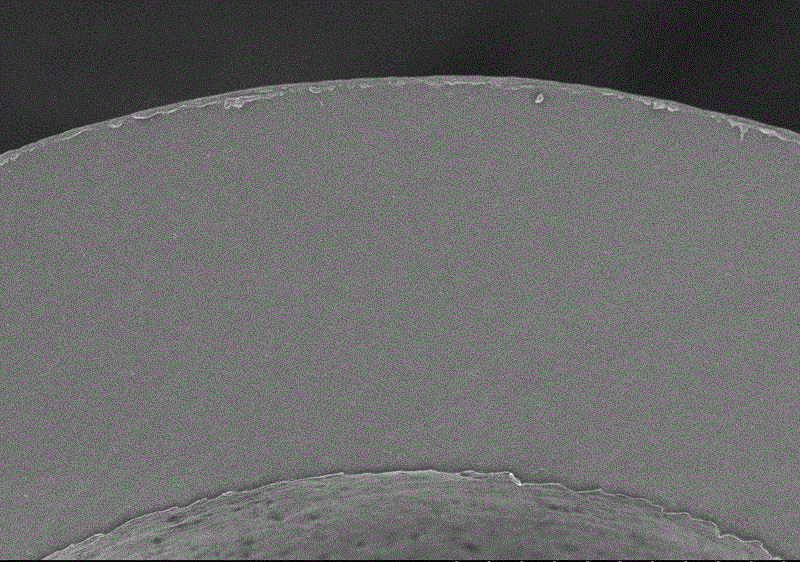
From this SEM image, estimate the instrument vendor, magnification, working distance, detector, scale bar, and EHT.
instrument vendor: Hitachi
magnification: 1.3 K X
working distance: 8.1 mm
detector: SE(M)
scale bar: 40000 nm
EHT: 3 kV